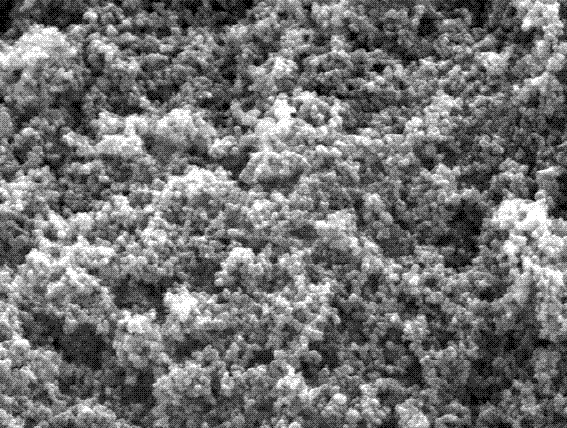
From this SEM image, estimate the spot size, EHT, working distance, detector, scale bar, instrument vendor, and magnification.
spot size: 3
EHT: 20 kV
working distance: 9.6 mm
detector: ETD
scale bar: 1000 nm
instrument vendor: FEI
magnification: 100 K X